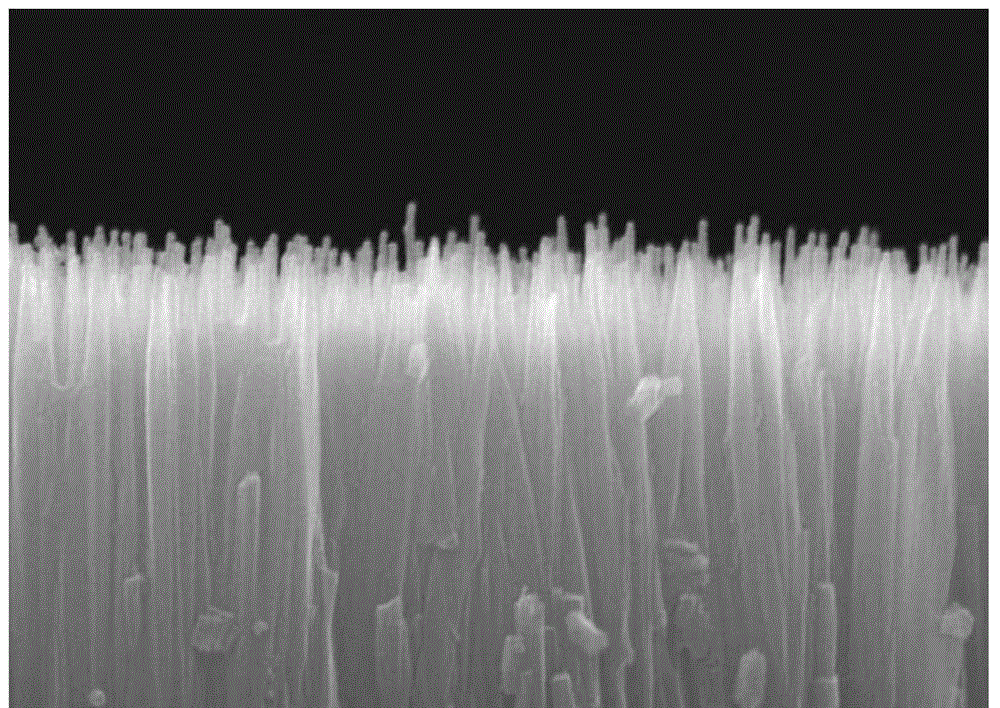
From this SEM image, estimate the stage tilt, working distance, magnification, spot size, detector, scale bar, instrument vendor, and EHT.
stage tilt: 0°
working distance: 9.1 mm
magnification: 100 K X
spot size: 3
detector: ETD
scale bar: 500 nm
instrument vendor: FEI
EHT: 30 kV